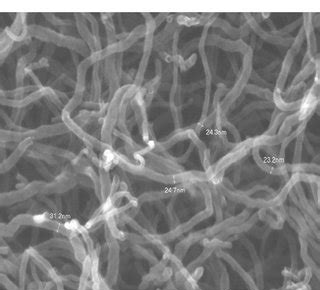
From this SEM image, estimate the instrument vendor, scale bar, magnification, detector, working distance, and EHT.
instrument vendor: JEOL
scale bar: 100 nm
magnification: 100 K X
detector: SEI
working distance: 8.6 mm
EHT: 15 kV